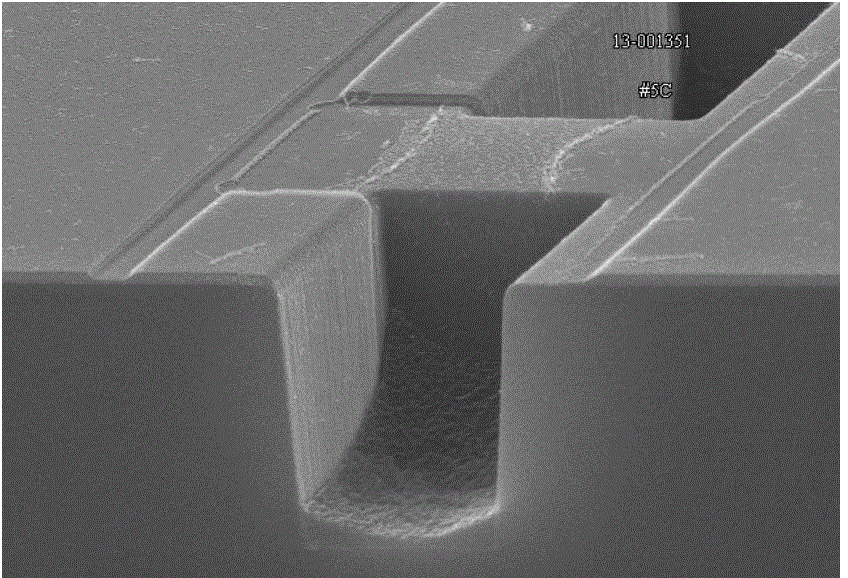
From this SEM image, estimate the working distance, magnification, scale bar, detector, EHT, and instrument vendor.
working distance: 8.6 mm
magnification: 13 K X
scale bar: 4000 nm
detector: SE(U)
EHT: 15 kV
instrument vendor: Hitachi